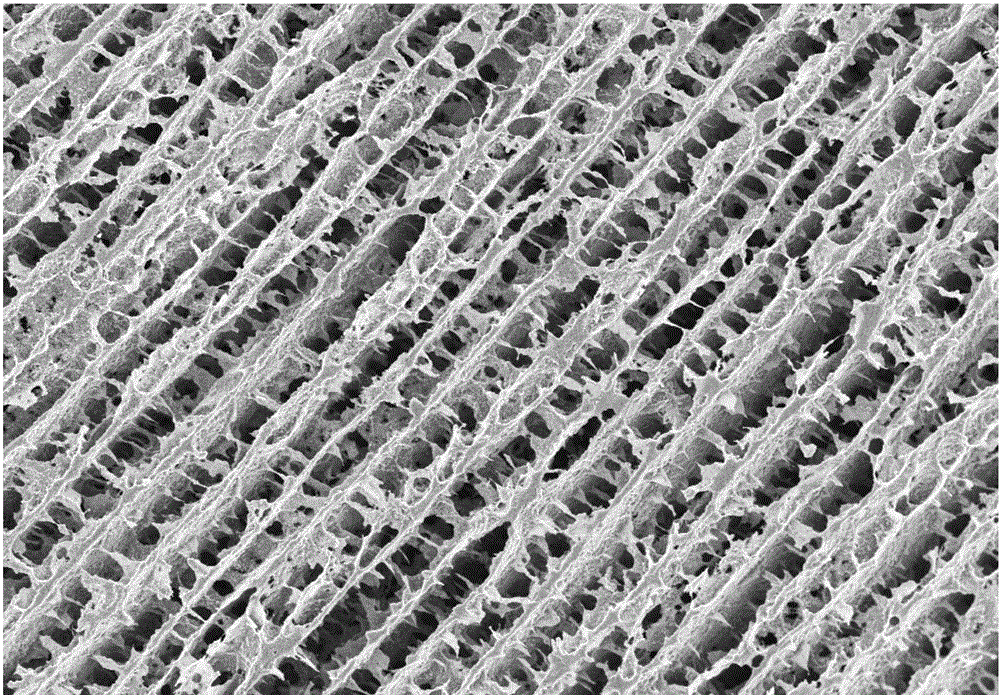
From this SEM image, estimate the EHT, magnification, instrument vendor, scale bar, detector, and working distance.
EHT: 5 kV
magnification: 0.3 K X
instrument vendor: Zeiss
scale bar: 20000 nm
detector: InLens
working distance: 7.3 mm